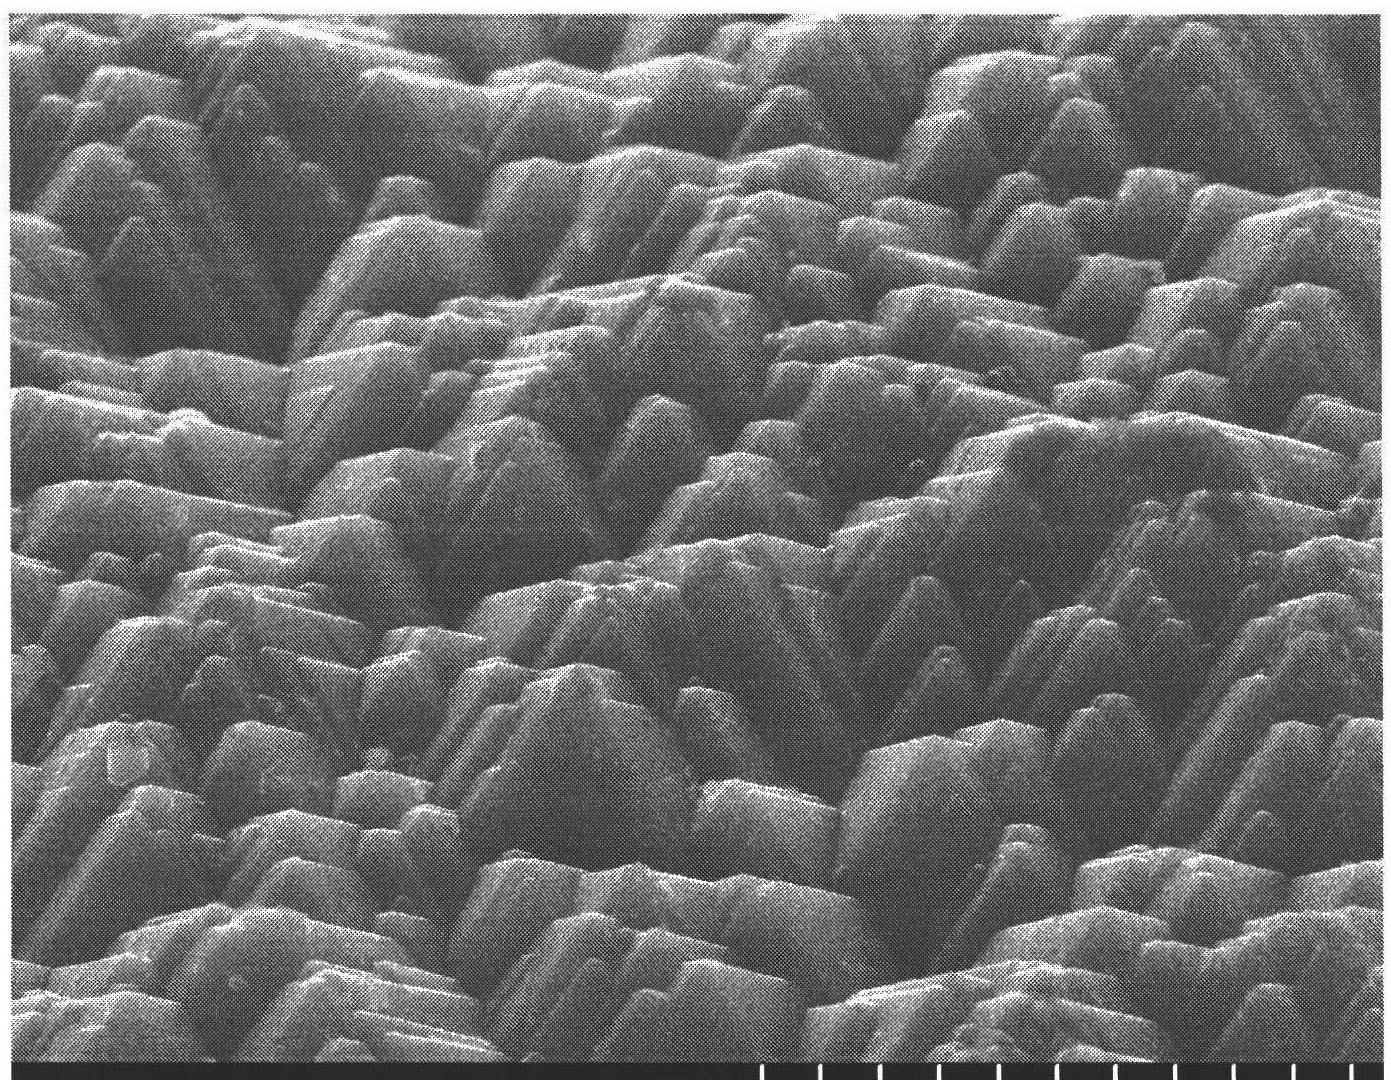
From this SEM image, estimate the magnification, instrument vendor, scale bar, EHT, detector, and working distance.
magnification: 5 K X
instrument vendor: Hitachi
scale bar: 10000 nm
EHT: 15 kV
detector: SE(U)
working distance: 10 mm